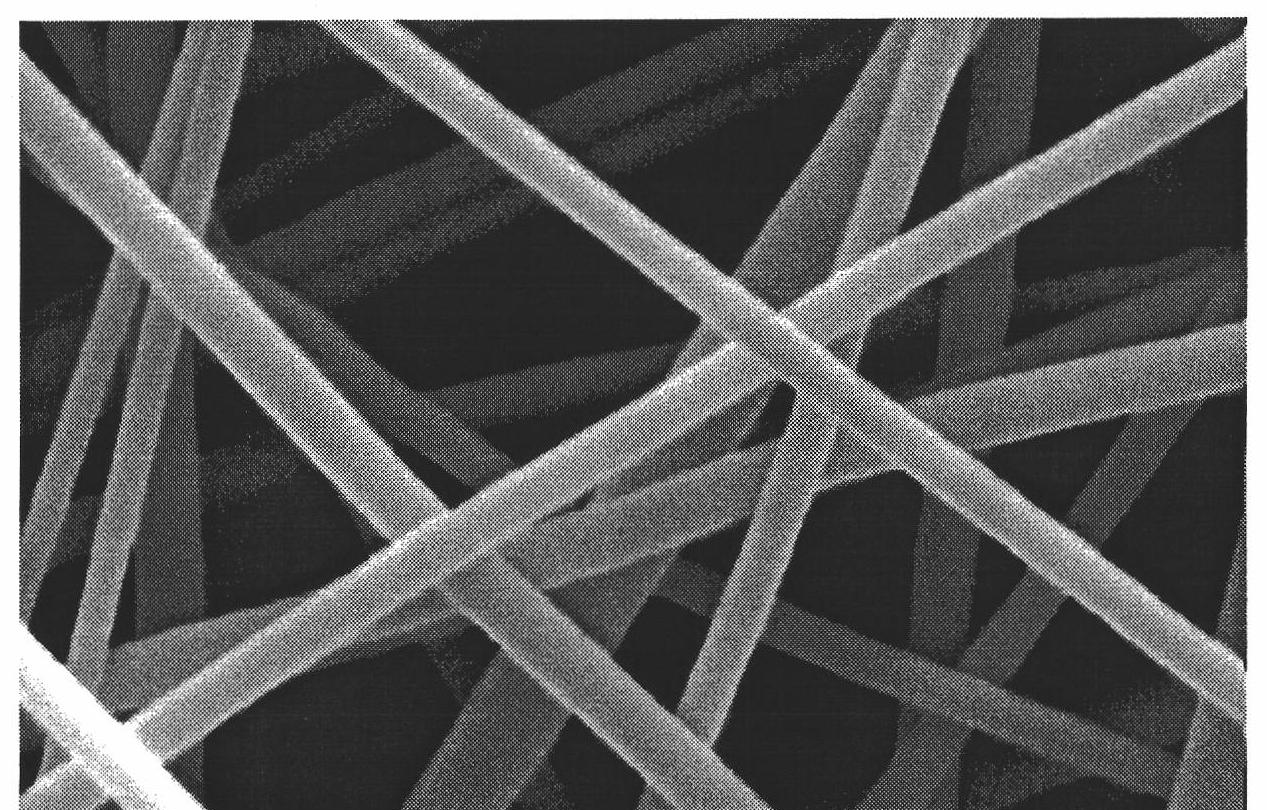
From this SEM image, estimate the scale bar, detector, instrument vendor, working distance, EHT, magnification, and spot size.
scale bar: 1000 nm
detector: SE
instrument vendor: FEI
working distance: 7.5 mm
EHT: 5 kV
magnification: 50 K X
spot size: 3